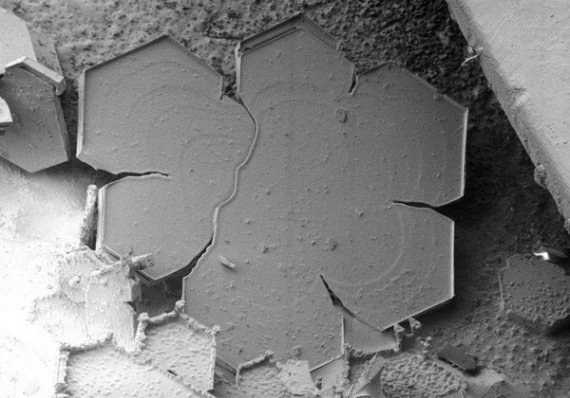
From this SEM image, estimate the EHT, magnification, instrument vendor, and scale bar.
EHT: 2 kV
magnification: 0.04 K X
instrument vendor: JEOL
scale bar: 750000 nm